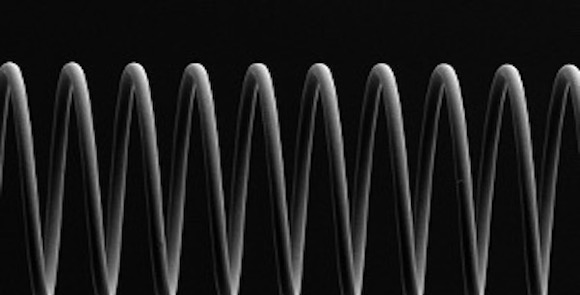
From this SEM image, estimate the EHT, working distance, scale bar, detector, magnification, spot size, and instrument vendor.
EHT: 15 kV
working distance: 11 mm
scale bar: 100000 nm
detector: SEI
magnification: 0.17 K X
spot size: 40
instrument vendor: JEOL